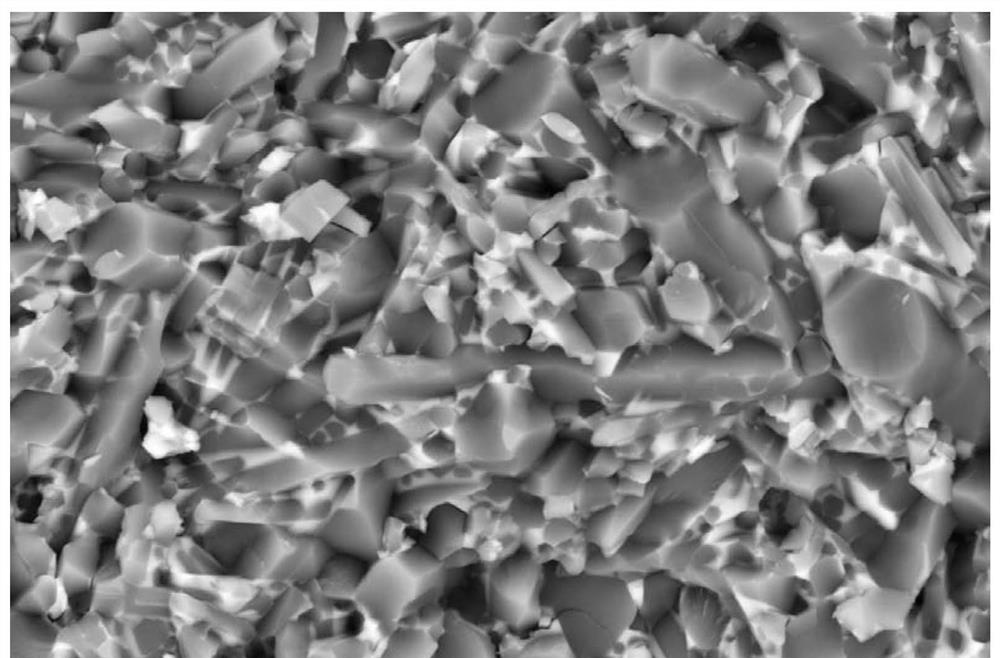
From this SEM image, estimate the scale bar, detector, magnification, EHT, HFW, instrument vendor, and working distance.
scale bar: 5000 nm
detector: CBS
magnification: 8 K X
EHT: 10 kV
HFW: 15.9 µm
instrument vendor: Thermo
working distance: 4.5 mm